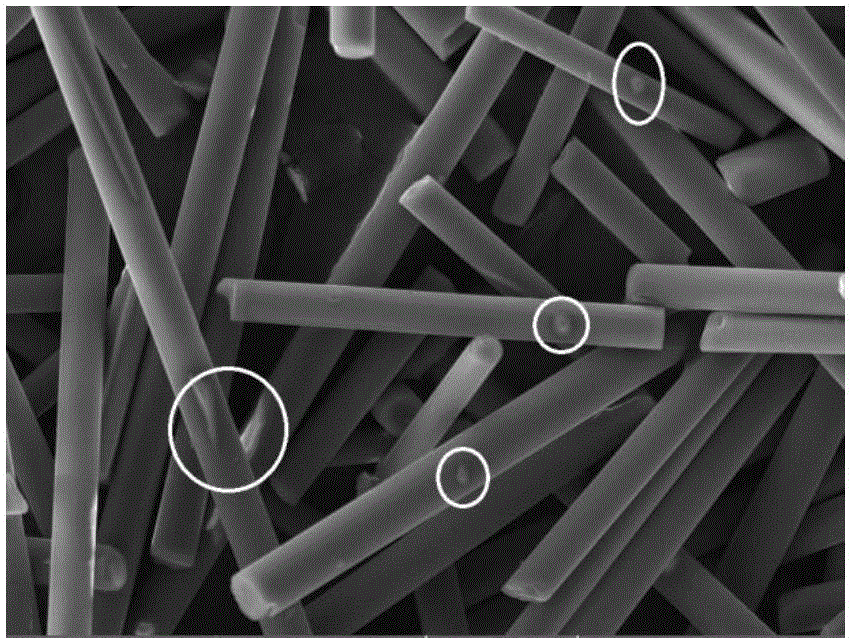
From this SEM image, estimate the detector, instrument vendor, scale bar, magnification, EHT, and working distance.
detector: SE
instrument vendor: Tescan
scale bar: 50000 nm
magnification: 1 K X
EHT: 30 kV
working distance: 9.05 mm